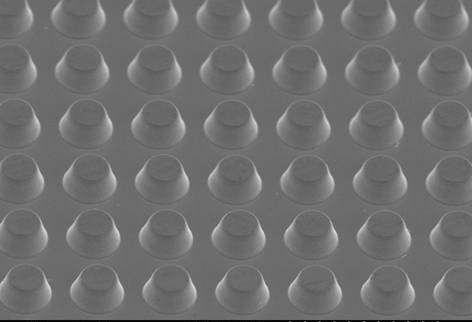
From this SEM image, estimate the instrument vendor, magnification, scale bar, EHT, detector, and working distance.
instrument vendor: Hitachi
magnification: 2 K X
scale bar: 20000 nm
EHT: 5 kV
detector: SE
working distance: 10.5 mm